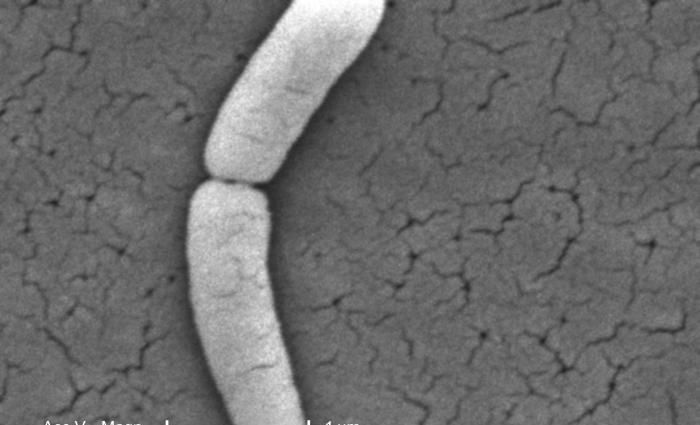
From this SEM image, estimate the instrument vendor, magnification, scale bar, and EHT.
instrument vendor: FEI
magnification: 25 K X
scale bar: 1000 nm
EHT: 30 kV